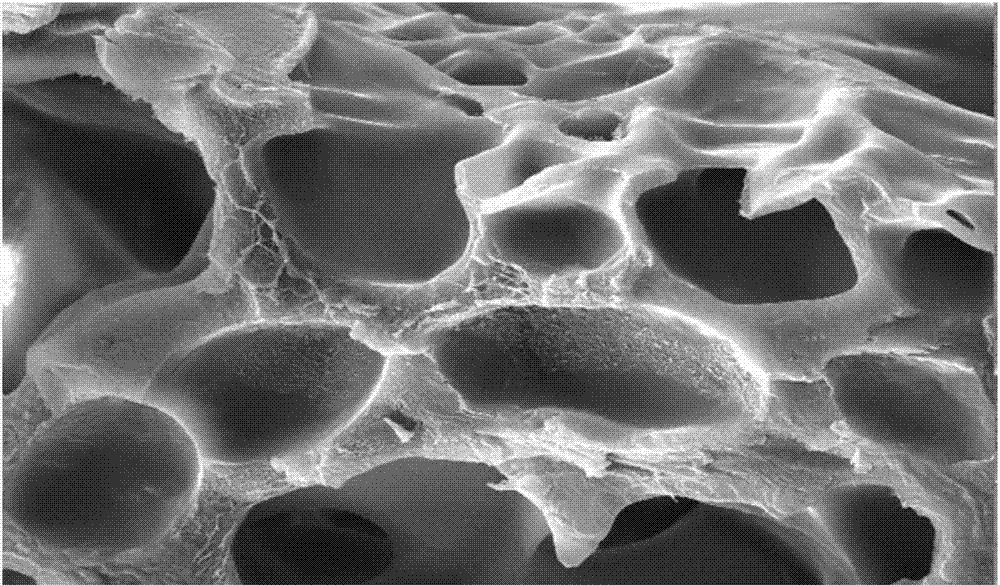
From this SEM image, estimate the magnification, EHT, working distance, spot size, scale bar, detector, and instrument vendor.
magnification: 1 K X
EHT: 20 kV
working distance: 9.8 mm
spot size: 5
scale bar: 20000 nm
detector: SE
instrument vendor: FEI